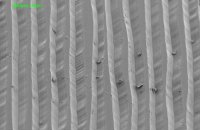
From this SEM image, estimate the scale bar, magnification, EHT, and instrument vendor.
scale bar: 100000 nm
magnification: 0.12 K X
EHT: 10 kV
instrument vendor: JEOL